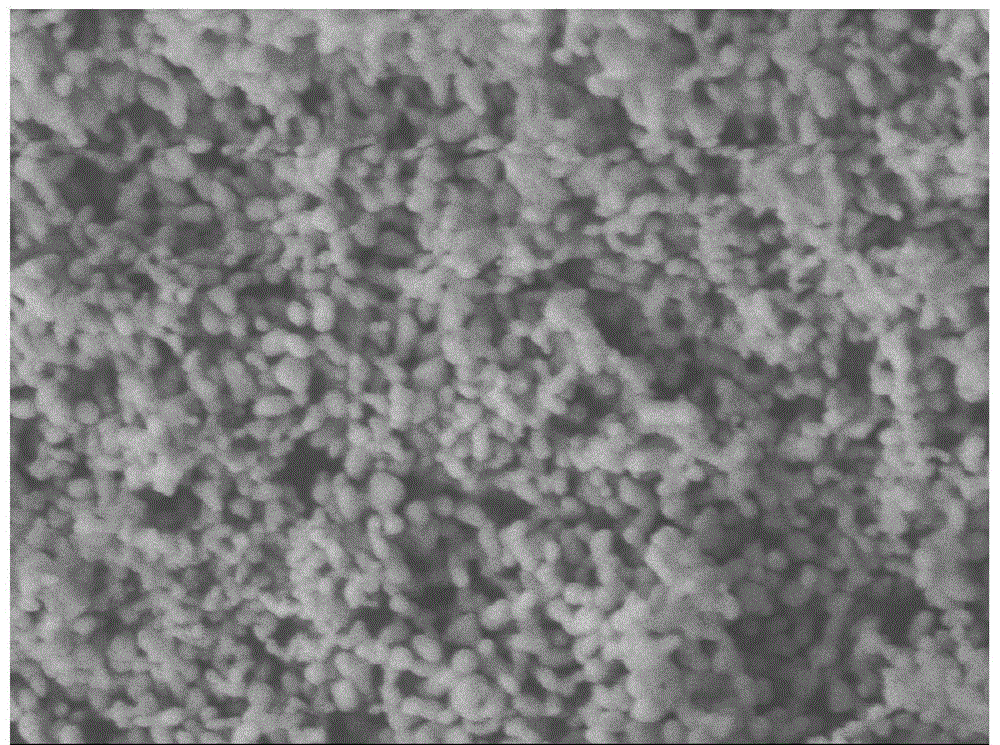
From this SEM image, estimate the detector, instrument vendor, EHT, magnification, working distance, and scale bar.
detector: LEI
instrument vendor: JEOL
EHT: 5 kV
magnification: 30 K X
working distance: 9 mm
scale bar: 100 nm